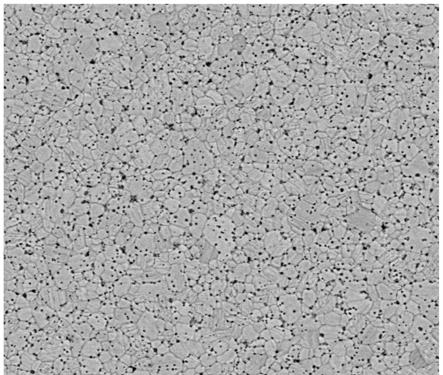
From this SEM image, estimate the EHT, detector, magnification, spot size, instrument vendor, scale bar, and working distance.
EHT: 20 kV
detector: BSED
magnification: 2 K X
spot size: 3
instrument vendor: FEI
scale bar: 50000 nm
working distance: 11.2 mm